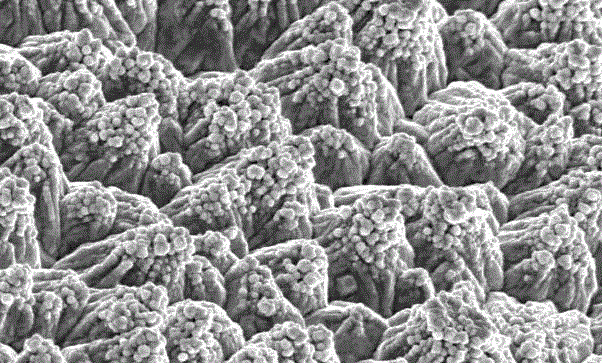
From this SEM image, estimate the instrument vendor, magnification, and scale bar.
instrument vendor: Hitachi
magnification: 1 K X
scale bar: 10000 nm